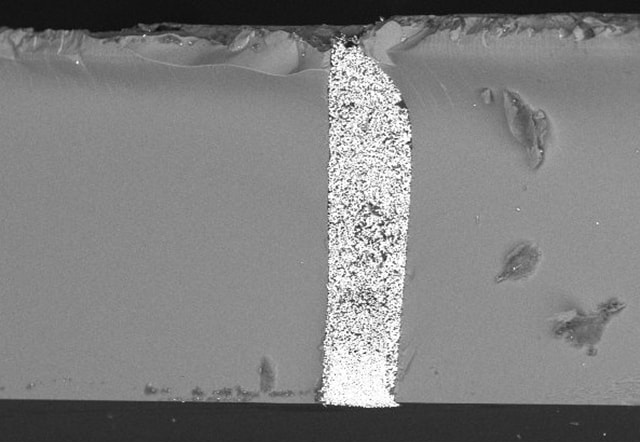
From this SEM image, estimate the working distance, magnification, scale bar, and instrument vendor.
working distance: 9.6 mm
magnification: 0.25 K X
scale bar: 300000 nm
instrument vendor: Hitachi